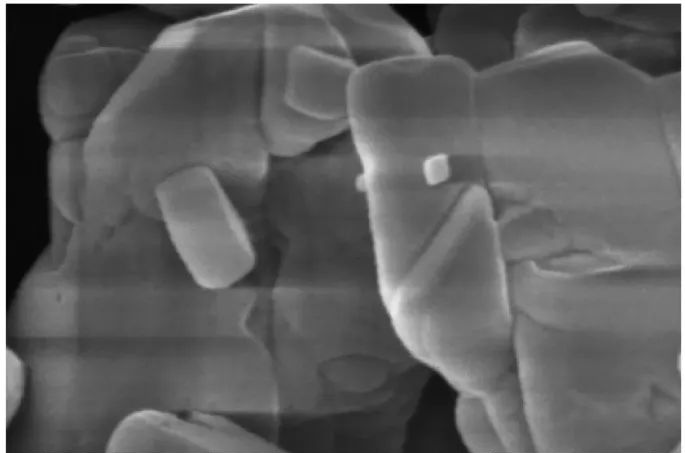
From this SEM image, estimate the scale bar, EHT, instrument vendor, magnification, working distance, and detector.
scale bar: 500 nm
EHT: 5 kV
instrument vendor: Thermo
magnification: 200 K X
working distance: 10 mm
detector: T2